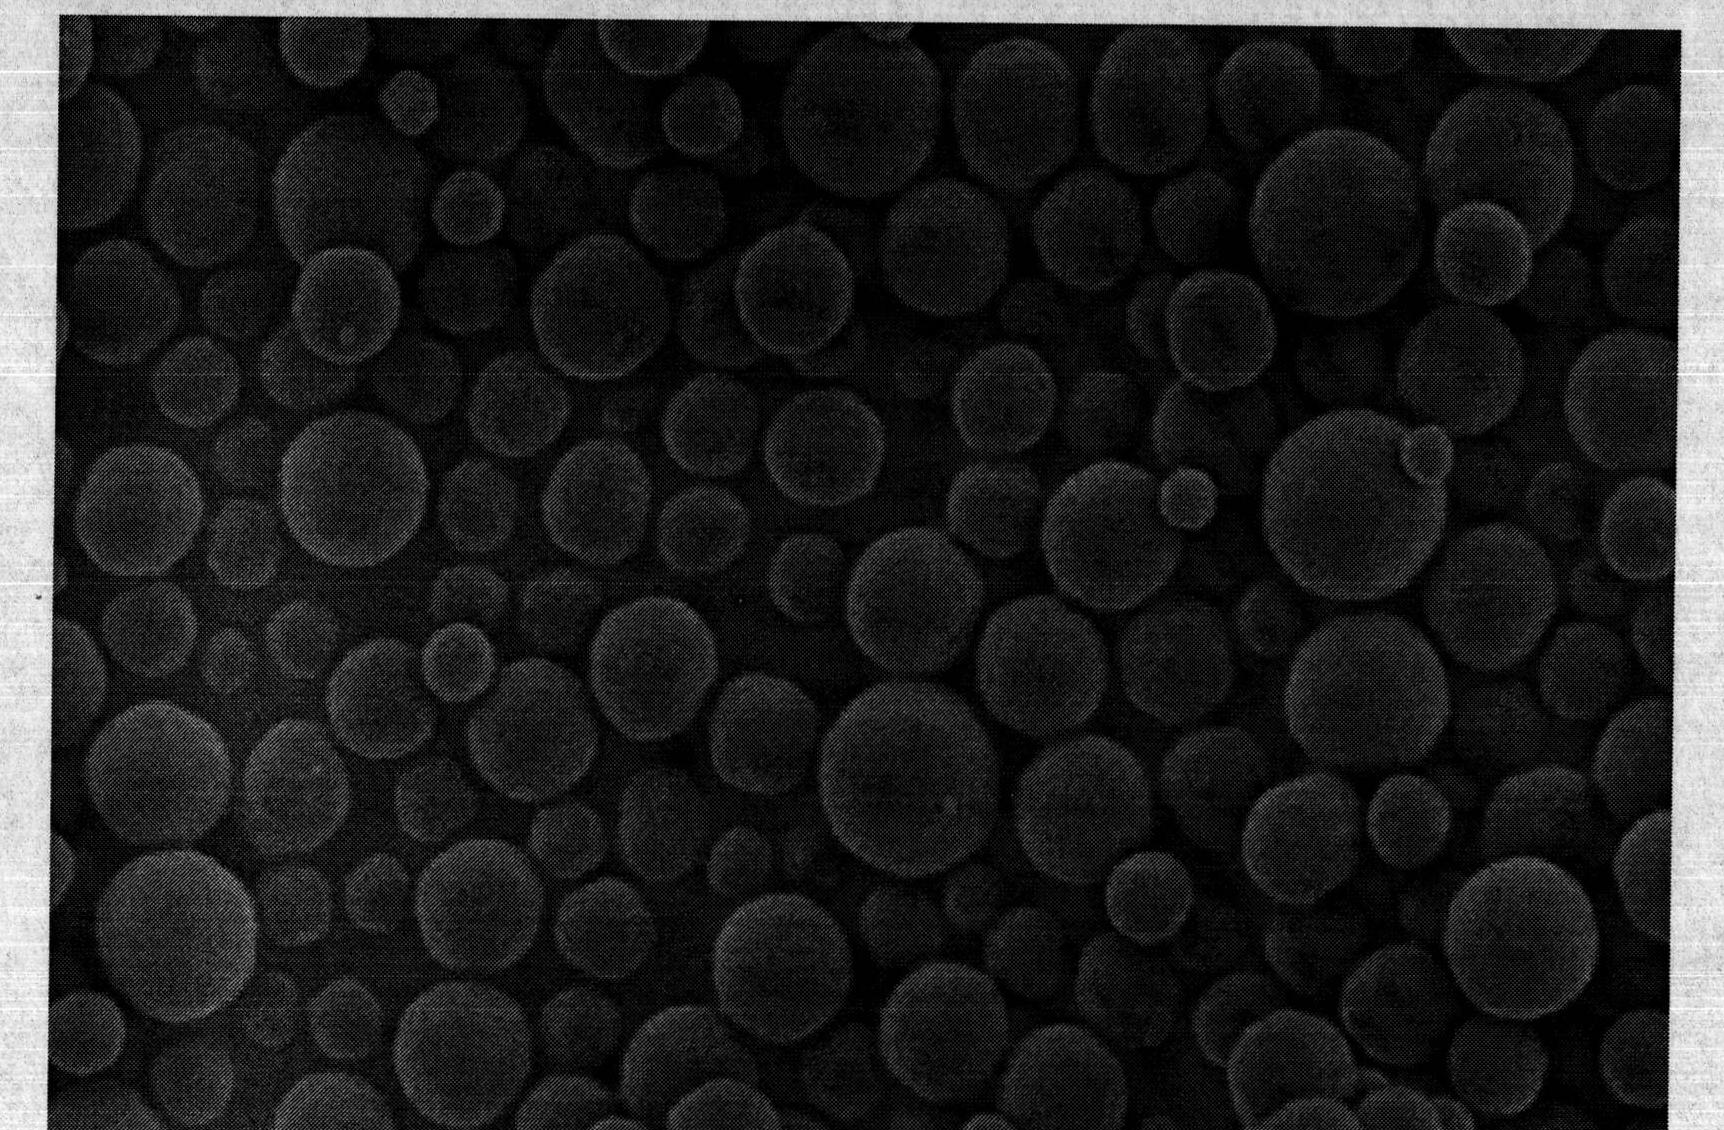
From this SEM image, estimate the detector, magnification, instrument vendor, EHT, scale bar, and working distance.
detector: SE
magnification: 5 K X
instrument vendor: Hitachi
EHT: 5 kV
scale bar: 10000 nm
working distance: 5.8 mm